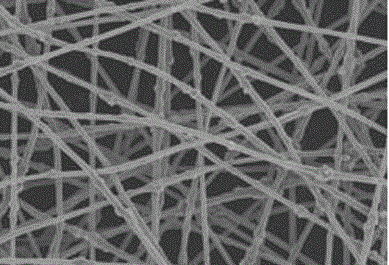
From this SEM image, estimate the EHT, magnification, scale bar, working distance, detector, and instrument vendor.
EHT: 5 kV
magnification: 4.5 K X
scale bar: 10000 nm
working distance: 9.3 mm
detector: SE(M)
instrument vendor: Hitachi